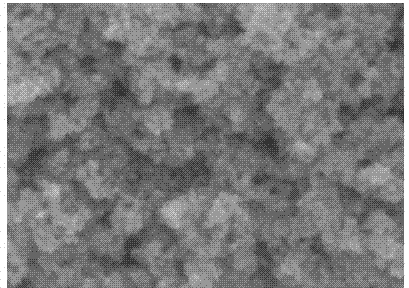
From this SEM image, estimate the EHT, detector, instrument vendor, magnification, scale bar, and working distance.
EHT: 5 kV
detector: SEI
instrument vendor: JEOL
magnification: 100 K X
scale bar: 100 nm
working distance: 9.2 mm